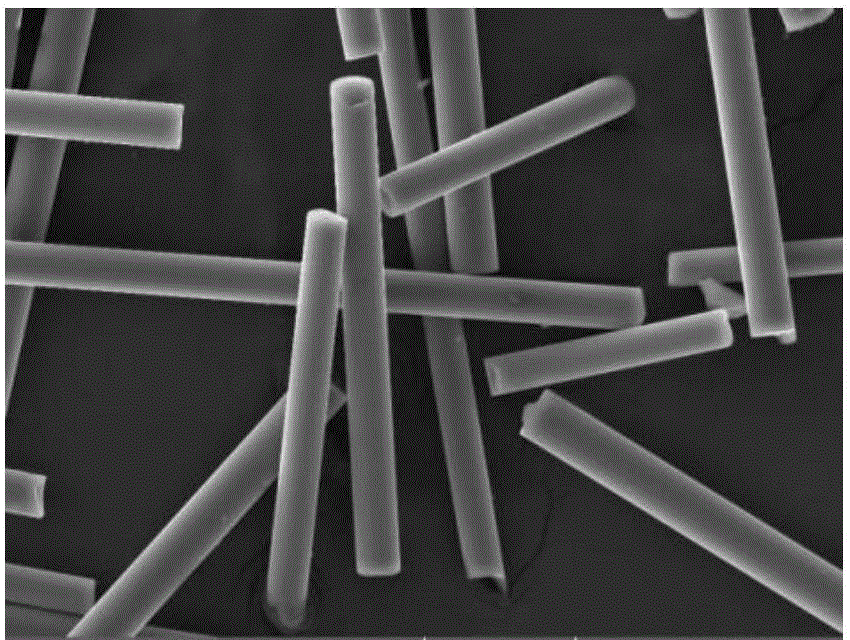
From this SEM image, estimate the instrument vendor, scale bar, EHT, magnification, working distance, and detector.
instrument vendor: Tescan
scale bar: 50000 nm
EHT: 30 kV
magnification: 1 K X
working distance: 9 mm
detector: SE